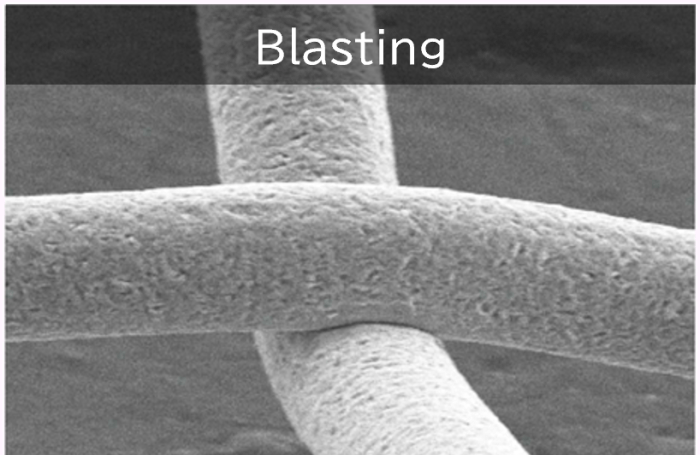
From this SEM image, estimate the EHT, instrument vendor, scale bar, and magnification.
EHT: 20 kV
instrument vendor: JEOL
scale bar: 10000 nm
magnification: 1.5 K X